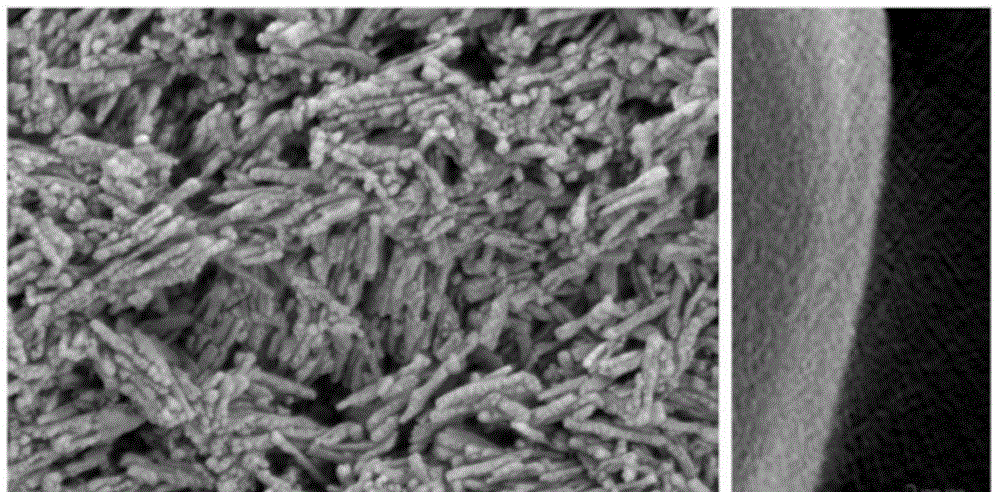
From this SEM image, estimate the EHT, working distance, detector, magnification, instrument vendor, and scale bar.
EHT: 3 kV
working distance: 7.4 mm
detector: SE2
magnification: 36.23 K X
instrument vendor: Zeiss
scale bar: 200 nm